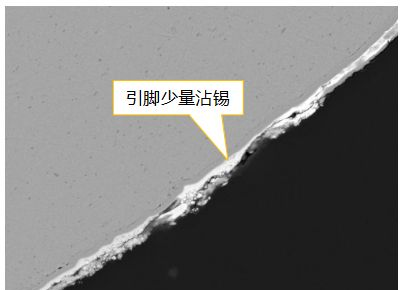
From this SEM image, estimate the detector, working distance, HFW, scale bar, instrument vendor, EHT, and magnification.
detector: BSE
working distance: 15.03 mm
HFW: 78.7 µm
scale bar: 20000 nm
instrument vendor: Tescan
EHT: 20 kV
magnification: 3.52 K X